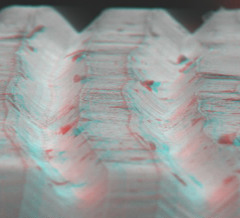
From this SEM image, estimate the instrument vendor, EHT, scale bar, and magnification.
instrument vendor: JEOL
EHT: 15 kV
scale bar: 100000 nm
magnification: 0.48 K X